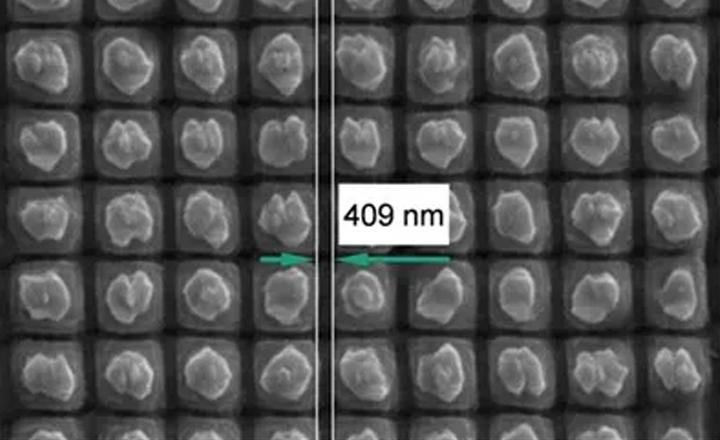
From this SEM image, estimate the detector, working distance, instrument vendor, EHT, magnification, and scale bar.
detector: SE1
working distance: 15 mm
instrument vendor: Zeiss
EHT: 20.56 kV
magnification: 20 K X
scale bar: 1000 nm